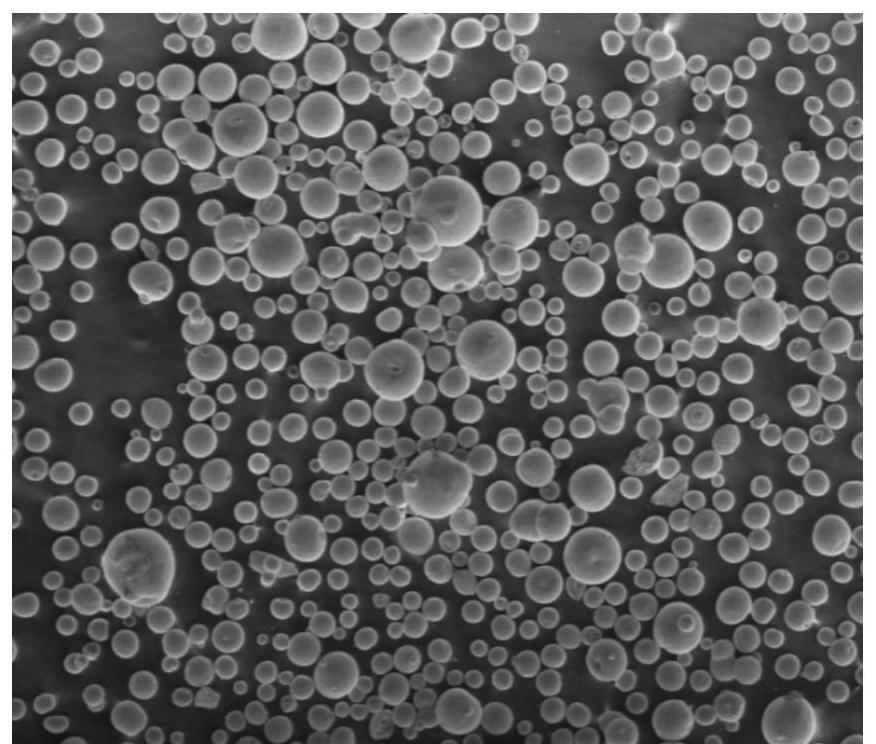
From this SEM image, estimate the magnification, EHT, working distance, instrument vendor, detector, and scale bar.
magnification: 0.15 K X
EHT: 3 kV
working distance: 6.5 mm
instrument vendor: FEI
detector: SE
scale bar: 500000 nm